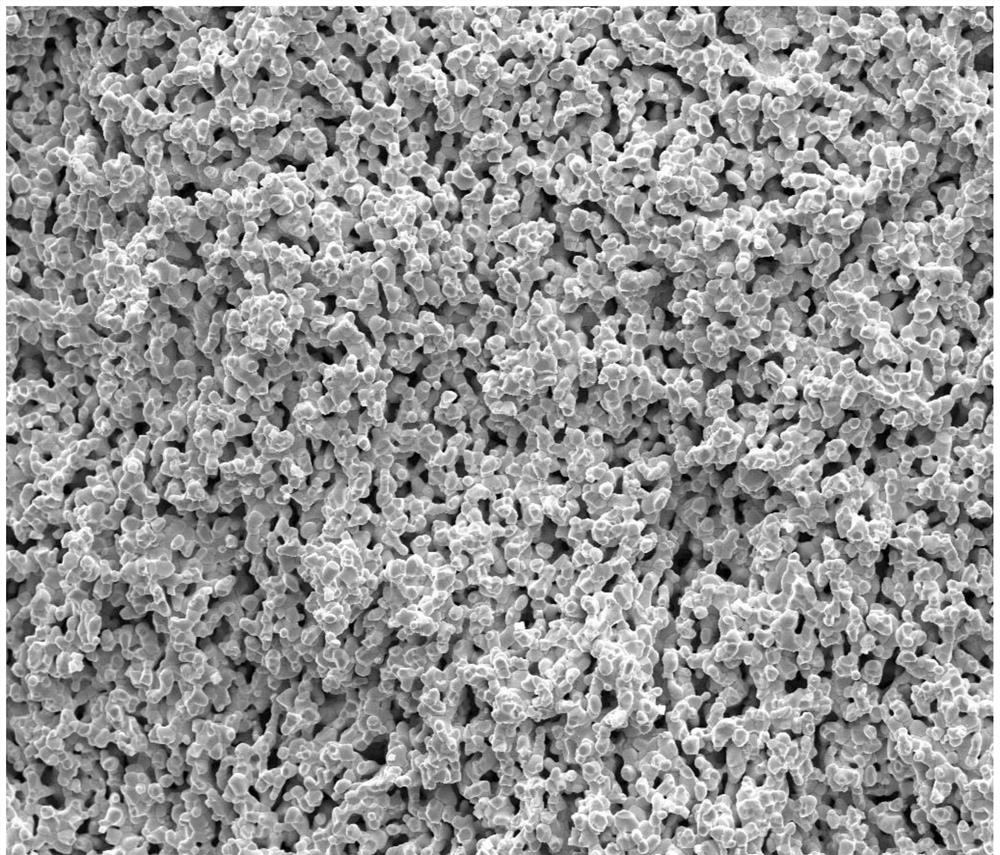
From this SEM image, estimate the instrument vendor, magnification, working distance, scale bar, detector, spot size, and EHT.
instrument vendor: FEI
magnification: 2 K X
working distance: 11 mm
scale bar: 50000 nm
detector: ETD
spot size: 3.5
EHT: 20 kV